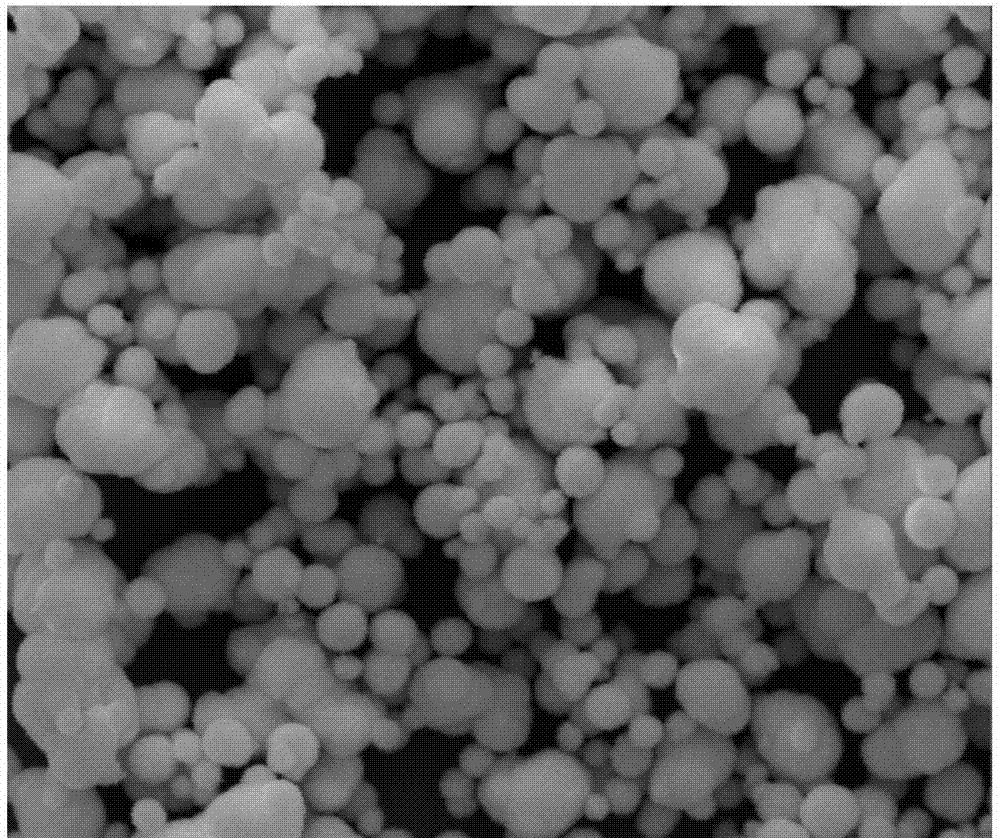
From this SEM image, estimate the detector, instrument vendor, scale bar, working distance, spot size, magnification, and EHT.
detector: ETD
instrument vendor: FEI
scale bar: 10000 nm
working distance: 8.7 mm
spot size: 3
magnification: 7 K X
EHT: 20 kV